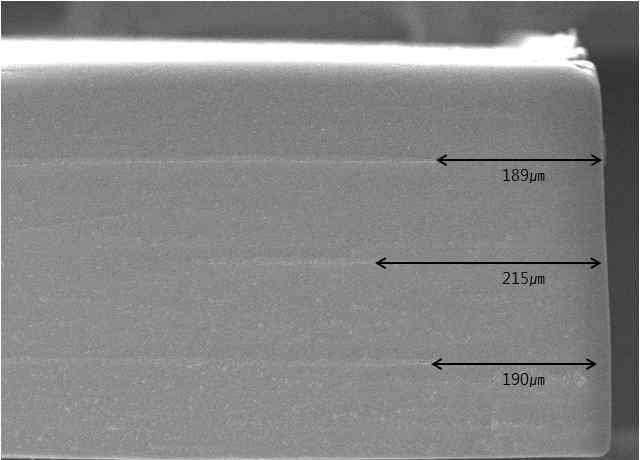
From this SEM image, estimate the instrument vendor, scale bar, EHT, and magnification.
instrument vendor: JEOL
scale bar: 200000 nm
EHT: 10 kV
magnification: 0.2 K X